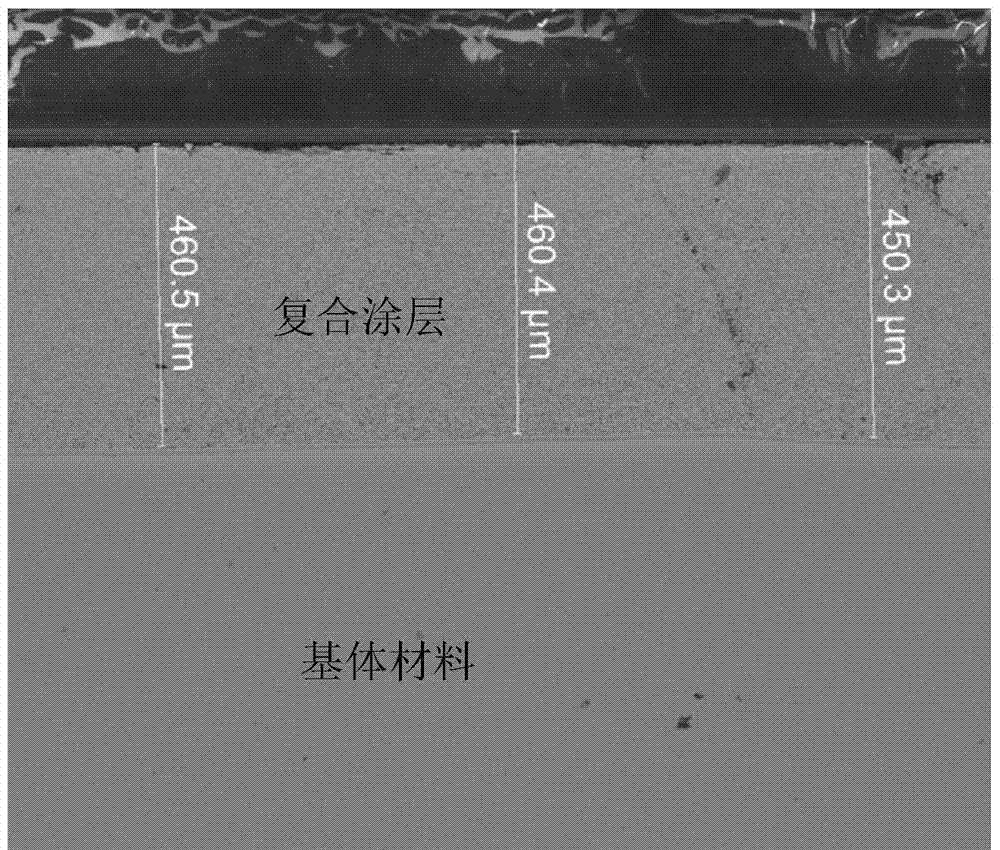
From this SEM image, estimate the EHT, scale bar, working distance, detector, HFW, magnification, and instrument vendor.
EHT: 20 kV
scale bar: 500000 nm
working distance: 11.6 mm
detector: BSED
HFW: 1490 µm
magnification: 0.2 K X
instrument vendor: FEI